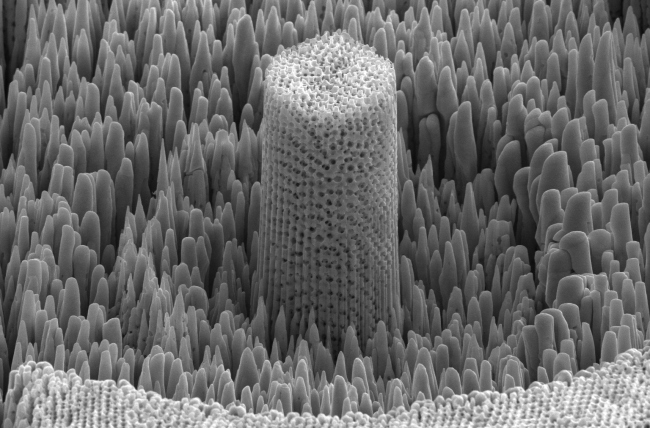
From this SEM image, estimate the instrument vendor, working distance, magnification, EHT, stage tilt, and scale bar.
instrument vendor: FEI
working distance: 4.1 mm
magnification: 11.97 K X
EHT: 10 kV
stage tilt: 52°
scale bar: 5000 nm